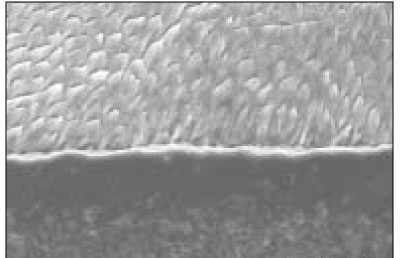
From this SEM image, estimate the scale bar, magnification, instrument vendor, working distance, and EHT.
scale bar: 50000 nm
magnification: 1 K X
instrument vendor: Hitachi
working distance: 12.6 mm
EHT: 15 kV